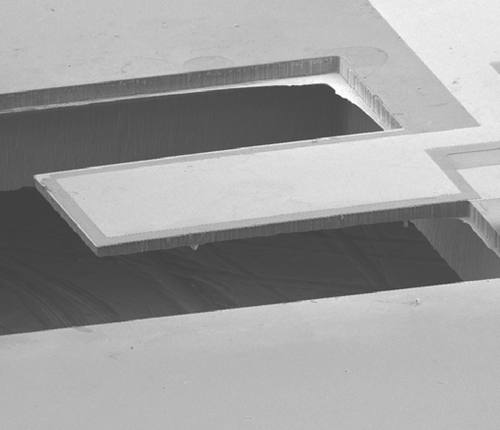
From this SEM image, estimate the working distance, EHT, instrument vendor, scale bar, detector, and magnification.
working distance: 30.9 mm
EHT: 10 kV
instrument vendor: JEOL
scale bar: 100000 nm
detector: SEI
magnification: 0.08 K X